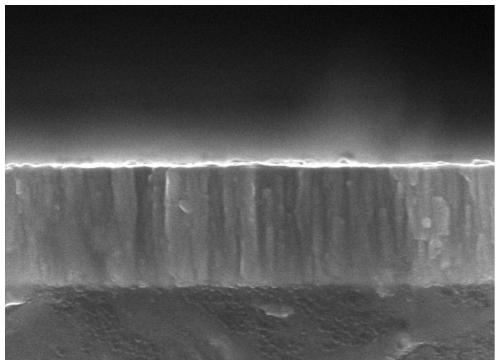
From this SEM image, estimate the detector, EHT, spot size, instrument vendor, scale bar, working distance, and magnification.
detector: TLD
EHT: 10 kV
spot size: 3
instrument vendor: FEI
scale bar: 500 nm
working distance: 6.1 mm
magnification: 50 K X